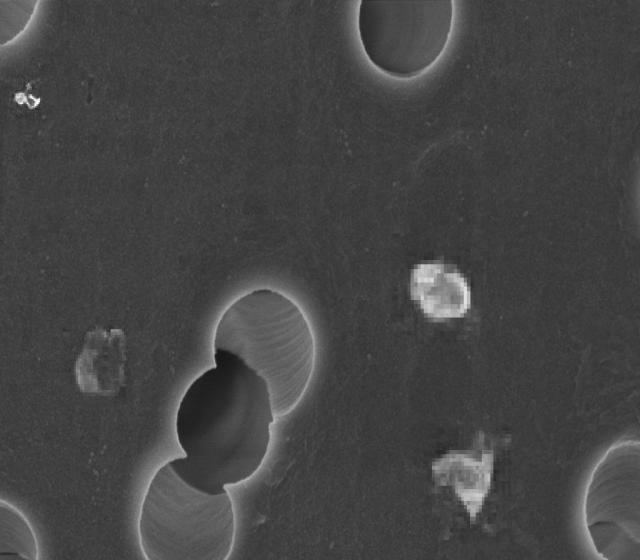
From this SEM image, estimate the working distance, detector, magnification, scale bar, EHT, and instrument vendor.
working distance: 2.9 mm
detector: InLens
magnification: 65 K X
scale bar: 200 nm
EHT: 3 kV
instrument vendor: Zeiss